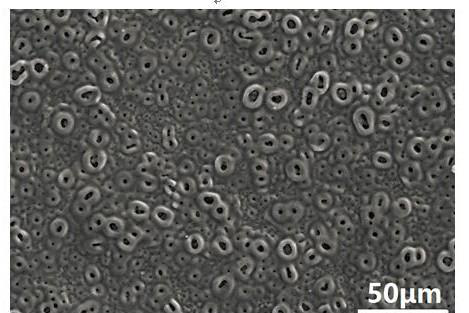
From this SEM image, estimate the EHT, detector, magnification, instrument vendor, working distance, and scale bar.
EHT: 20 kV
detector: SE(UL)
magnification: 0.5 K X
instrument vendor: Hitachi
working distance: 15.1 mm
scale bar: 100000 nm